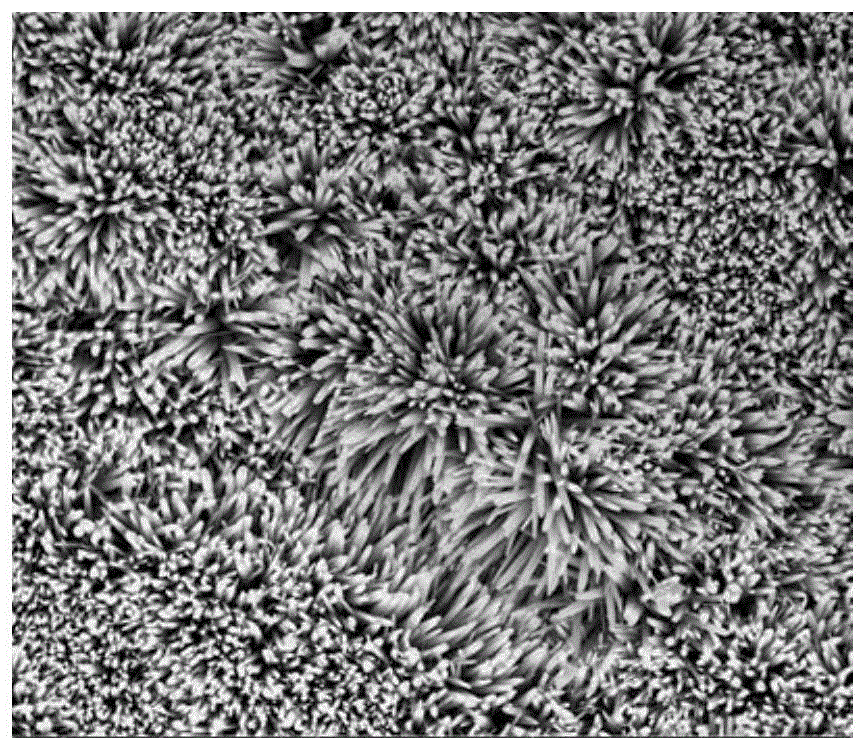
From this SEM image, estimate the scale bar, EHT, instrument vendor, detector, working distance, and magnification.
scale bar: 5000 nm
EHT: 15 kV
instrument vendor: FEI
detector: TLD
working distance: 5 mm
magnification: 10 K X